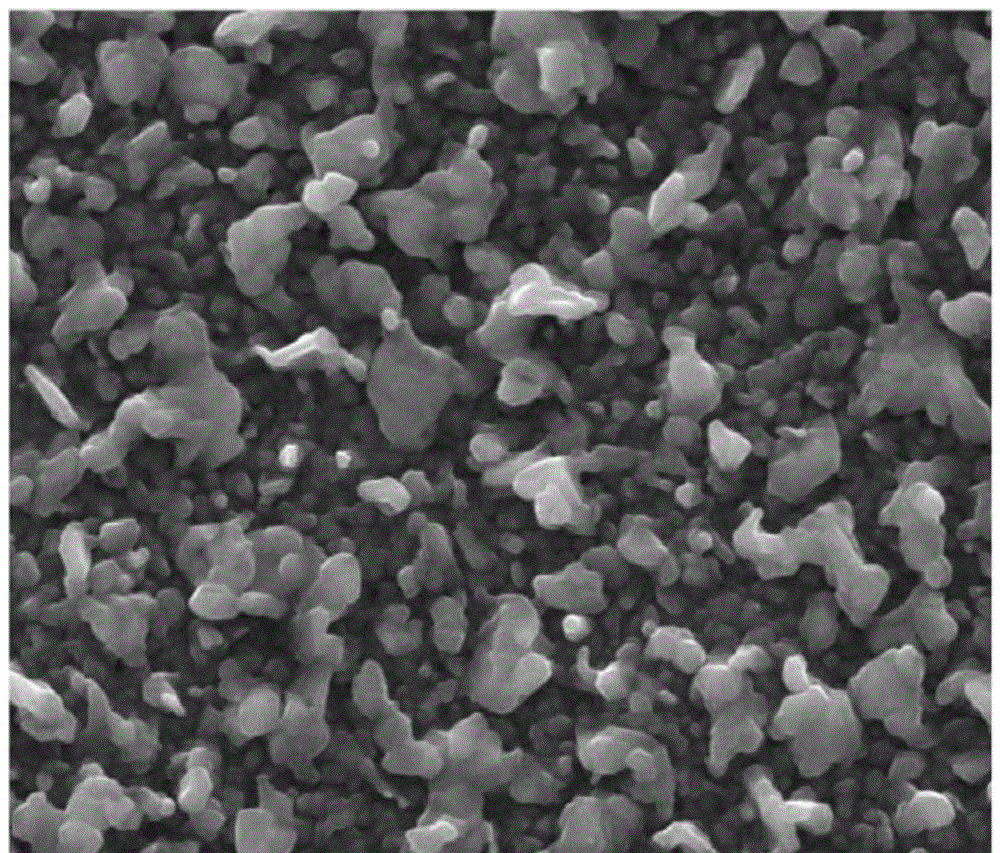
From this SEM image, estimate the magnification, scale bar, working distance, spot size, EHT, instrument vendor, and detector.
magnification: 20 K X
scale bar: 5000 nm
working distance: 9.8 mm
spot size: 3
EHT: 20 kV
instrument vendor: FEI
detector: ETD